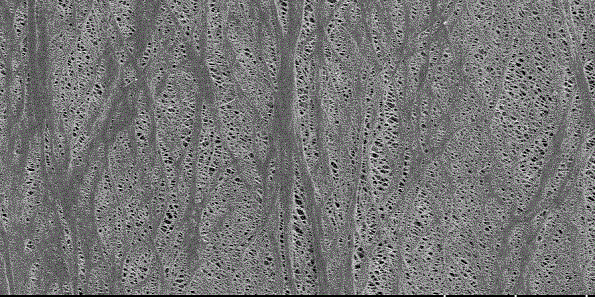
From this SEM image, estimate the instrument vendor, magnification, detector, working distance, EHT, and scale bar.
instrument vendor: Hitachi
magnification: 3 K X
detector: SE(UL)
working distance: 9.7 mm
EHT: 1 kV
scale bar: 10000 nm